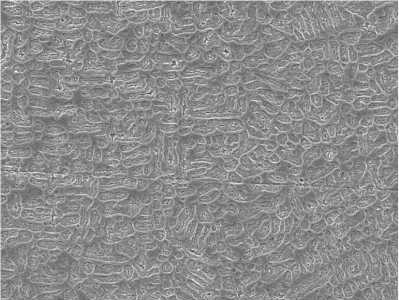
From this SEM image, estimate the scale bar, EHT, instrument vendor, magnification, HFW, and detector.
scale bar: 20000 nm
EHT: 20 kV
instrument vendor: Tescan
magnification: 2 K X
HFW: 150 µm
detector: SE Detector